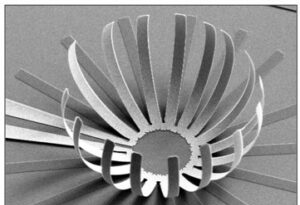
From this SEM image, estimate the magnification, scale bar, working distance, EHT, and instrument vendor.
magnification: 0.5 K X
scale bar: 50000 nm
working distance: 10 mm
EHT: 10 kV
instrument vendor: JEOL